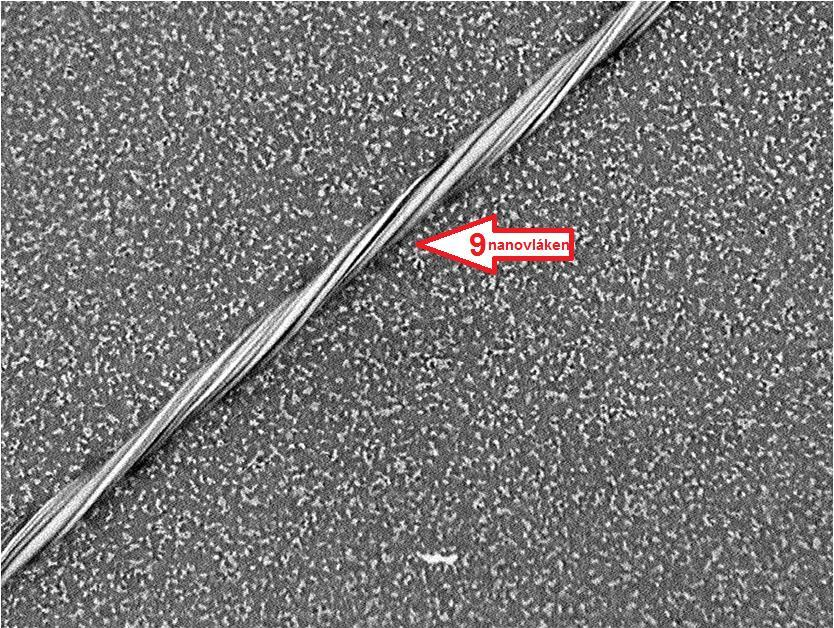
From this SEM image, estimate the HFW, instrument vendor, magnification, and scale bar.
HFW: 80 µm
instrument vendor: other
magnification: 3 K X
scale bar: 40000 nm